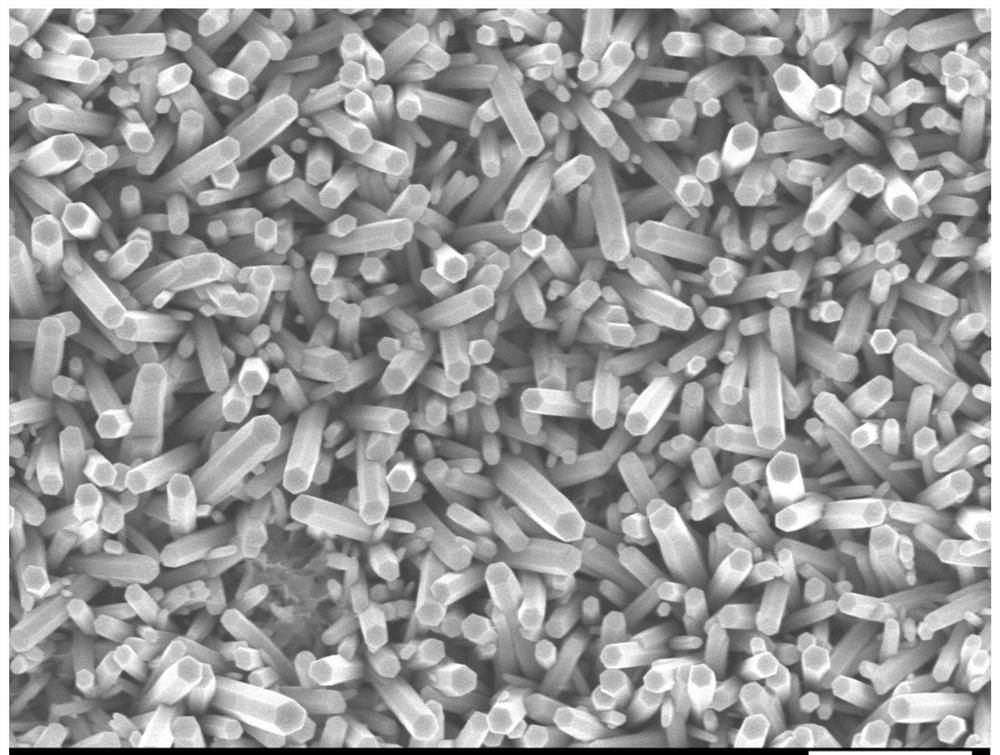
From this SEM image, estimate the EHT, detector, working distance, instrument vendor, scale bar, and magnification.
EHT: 5 kV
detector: SEI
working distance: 7 mm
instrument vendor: JEOL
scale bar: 1000 nm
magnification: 20 K X